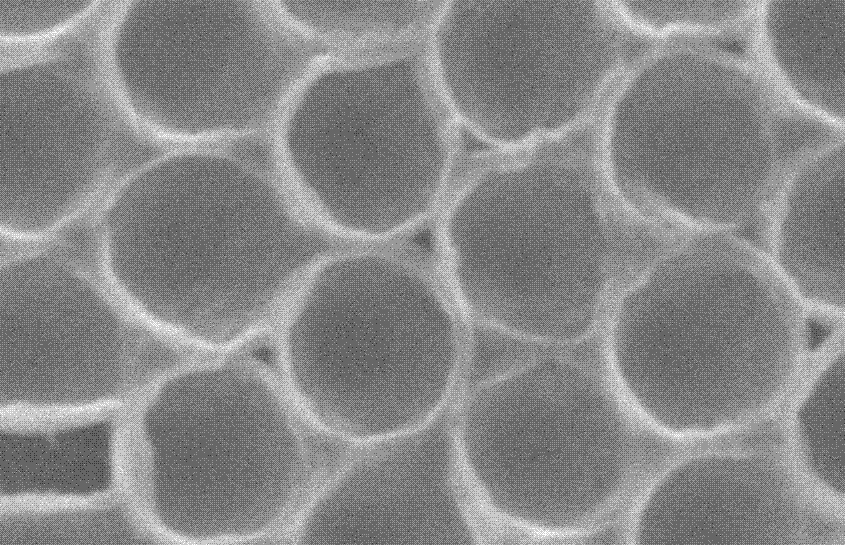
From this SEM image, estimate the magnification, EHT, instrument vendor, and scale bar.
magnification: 20 K X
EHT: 15 kV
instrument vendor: JEOL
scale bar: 1000 nm